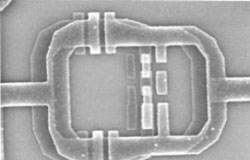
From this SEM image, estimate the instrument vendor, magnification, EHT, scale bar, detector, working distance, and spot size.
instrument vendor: FEI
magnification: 48.158 K X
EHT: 5 kV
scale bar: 1000 nm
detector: TLD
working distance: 5.3 mm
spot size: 3